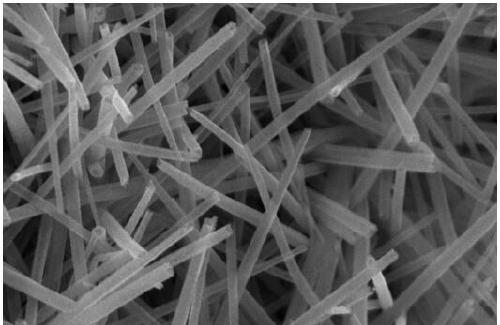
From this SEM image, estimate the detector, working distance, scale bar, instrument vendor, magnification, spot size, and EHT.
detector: TLD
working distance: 5.7 mm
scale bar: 1000 nm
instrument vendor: FEI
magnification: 100 K X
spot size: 3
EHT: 10 kV